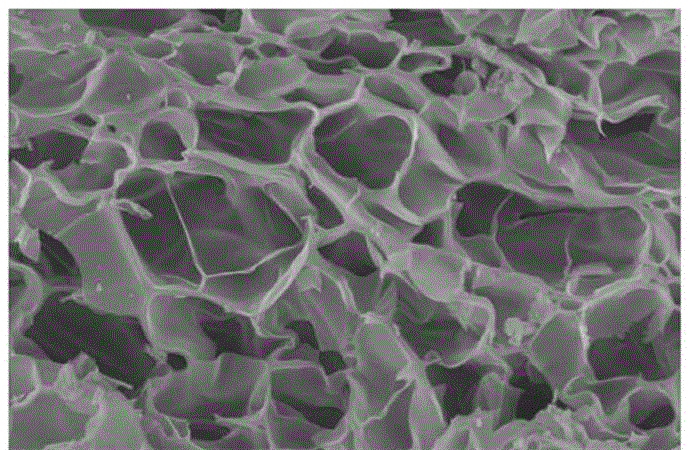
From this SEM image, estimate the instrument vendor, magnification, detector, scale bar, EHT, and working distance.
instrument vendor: Hitachi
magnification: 5 K X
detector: SE(M)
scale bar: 10000 nm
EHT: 15 kV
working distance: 9.7 mm